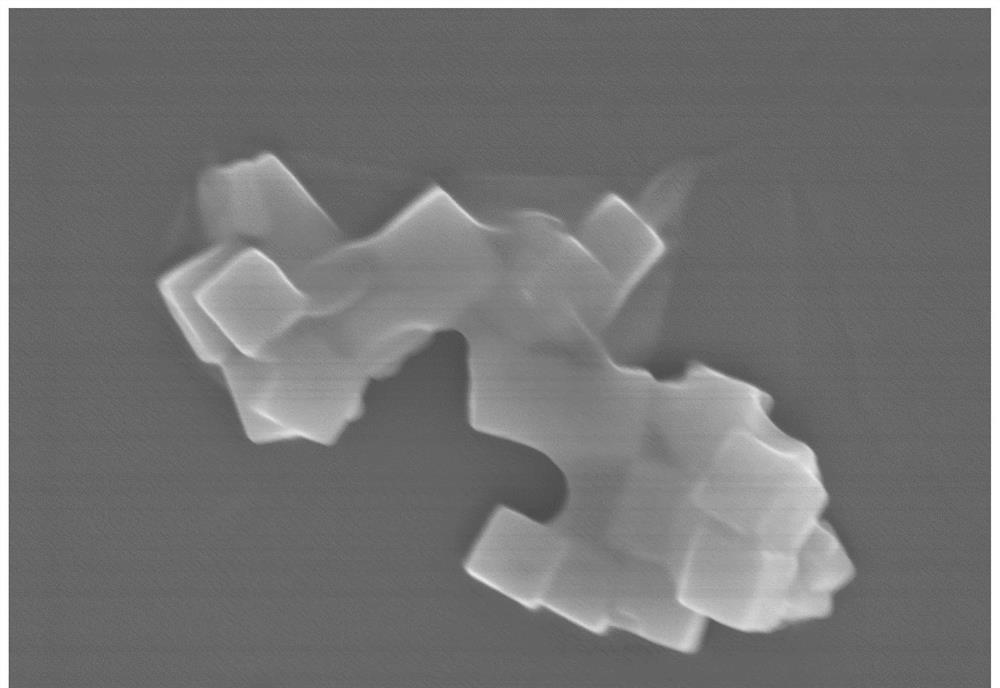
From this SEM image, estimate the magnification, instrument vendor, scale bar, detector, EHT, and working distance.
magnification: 30 K X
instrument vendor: Hitachi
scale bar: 1000 nm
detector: SE(UL)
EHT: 5 kV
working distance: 7.6 mm